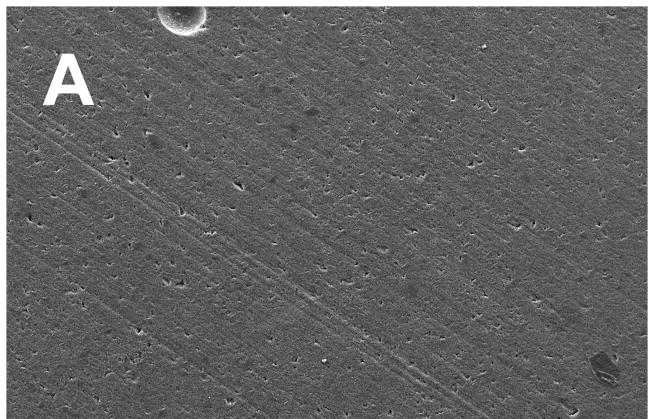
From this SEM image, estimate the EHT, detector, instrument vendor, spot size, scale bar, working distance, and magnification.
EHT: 15 kV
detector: SE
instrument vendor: FEI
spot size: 4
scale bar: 100000 nm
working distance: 9.9 mm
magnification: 0.2 K X